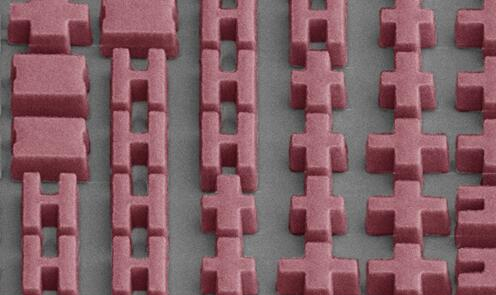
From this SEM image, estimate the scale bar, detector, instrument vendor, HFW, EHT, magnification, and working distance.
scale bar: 5000 nm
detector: ETD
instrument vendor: FEI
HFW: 16 µm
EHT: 5 kV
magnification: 8 K X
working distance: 4 mm